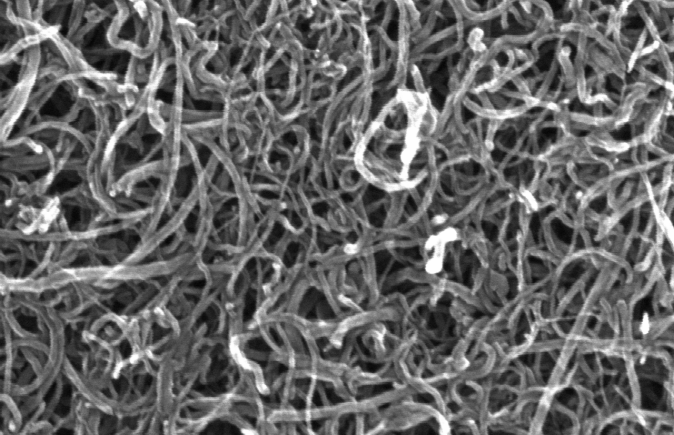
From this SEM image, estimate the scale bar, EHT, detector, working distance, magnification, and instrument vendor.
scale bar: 500 nm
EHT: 20 kV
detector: SE(M)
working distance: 7.8 mm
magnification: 100 K X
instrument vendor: Hitachi